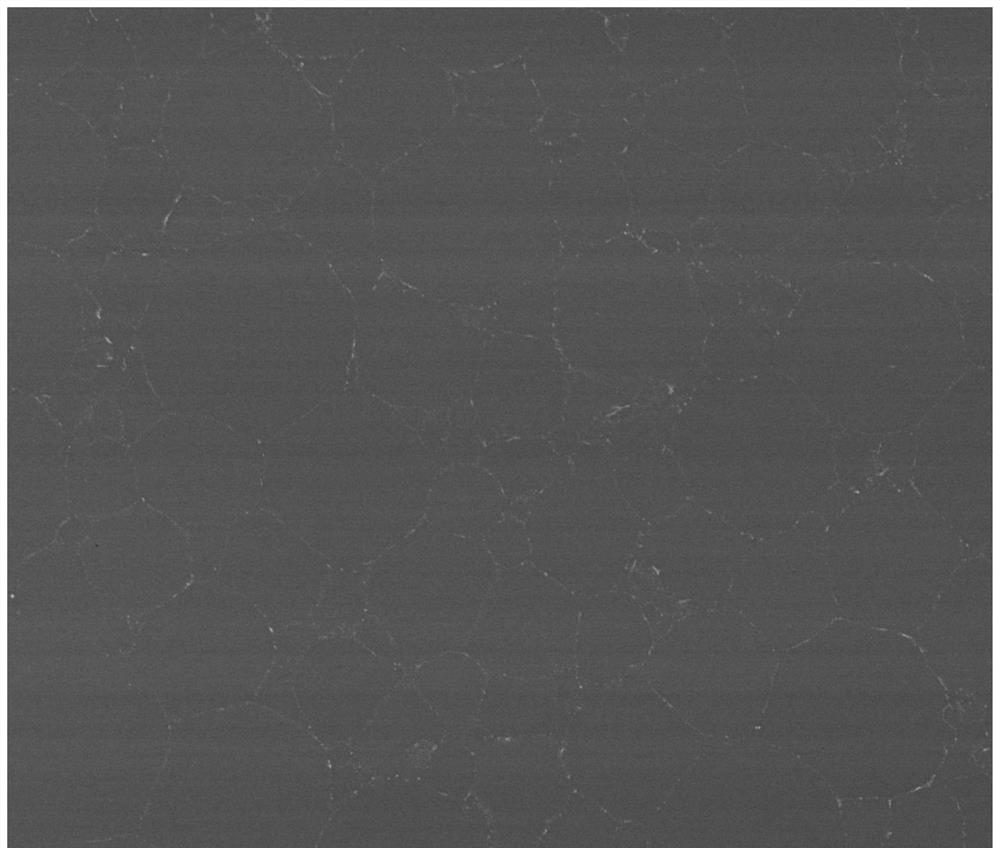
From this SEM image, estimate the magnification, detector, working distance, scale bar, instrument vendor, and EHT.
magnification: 1 K X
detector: Z Cont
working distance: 14.3 mm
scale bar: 100000 nm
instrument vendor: FEI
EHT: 30 kV